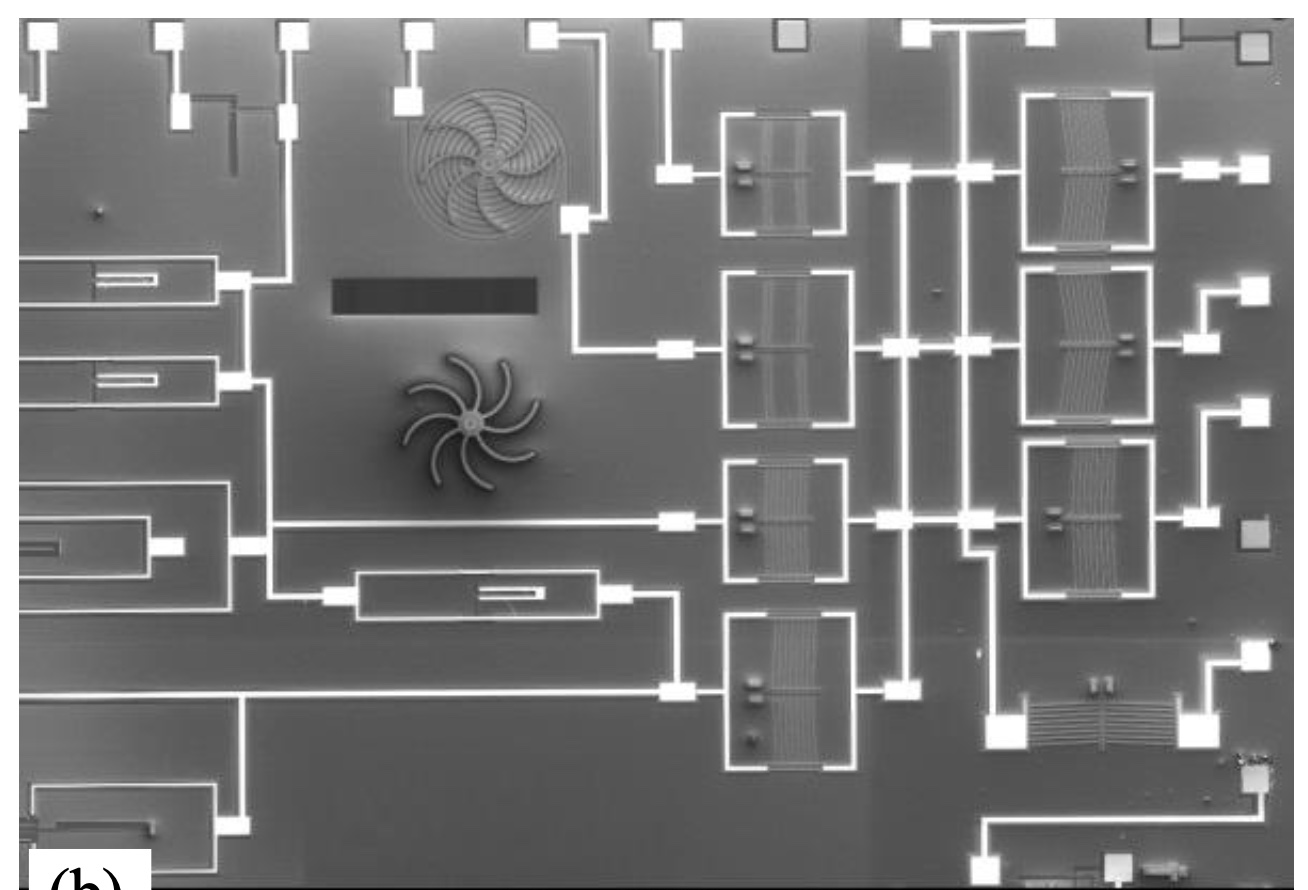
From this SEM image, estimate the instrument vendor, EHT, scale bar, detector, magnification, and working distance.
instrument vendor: JEOL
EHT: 5 kV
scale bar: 100000 nm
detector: SEI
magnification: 0.033 K X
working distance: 39.9 mm